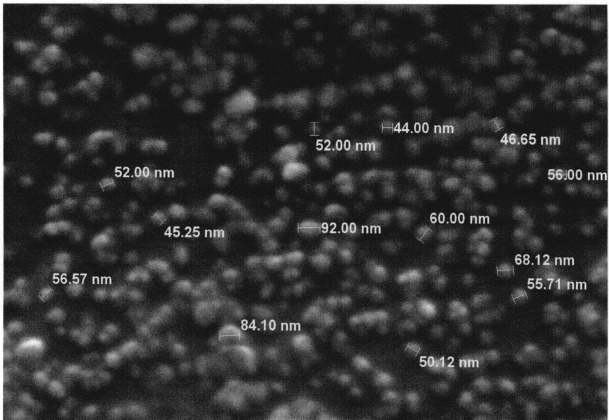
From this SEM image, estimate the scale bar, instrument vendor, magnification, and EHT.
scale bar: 500 nm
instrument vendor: JEOL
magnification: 50 K X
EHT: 15 kV